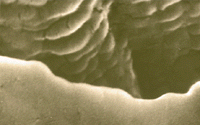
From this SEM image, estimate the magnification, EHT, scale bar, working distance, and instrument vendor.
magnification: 110 K X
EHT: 15 kV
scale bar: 100 nm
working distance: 14 mm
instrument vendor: JEOL